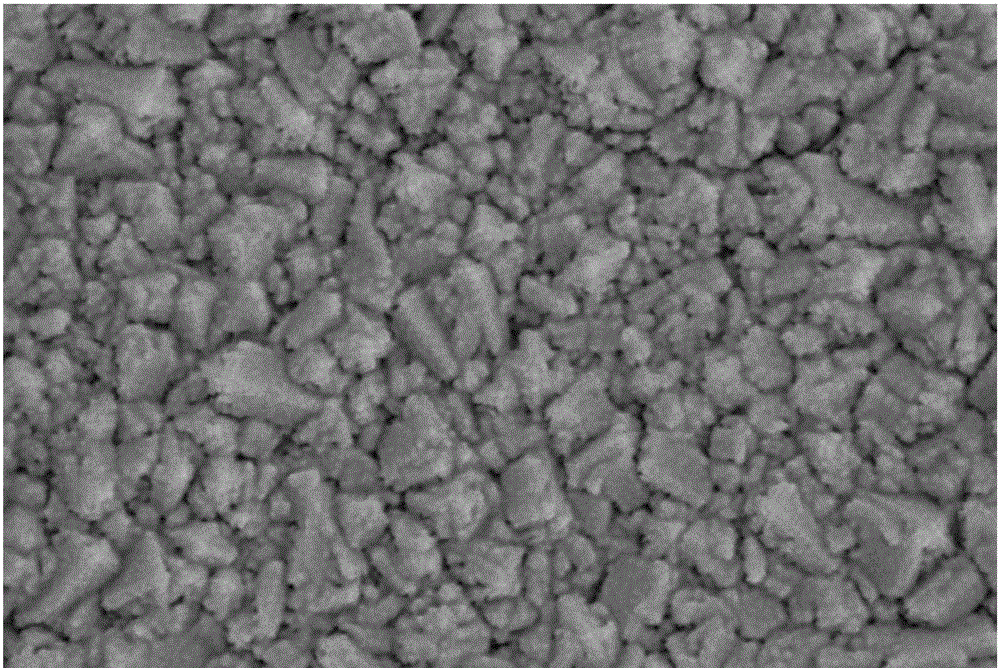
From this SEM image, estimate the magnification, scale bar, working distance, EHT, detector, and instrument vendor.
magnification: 50 K X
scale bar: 200 nm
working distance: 8.5 mm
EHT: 5 kV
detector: SE2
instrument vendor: Zeiss